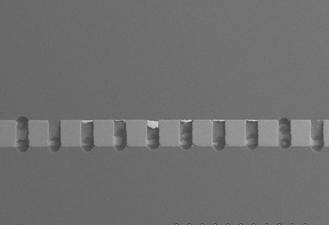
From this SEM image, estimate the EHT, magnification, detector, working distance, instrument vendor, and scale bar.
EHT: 15 kV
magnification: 0.05 K X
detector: BSE2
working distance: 14.7 mm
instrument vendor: JEOL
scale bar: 1000000 nm